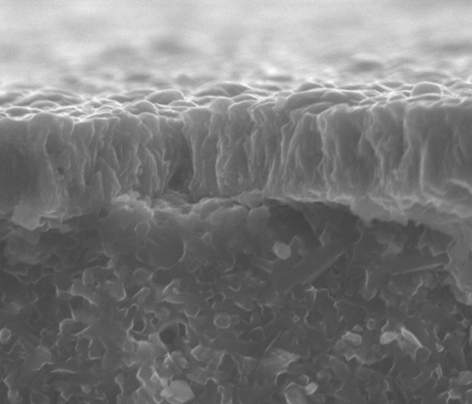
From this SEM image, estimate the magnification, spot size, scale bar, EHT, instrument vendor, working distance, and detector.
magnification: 6 K X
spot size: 4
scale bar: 10000 nm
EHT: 21.3 kV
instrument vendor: FEI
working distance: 11.5 mm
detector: GBSD